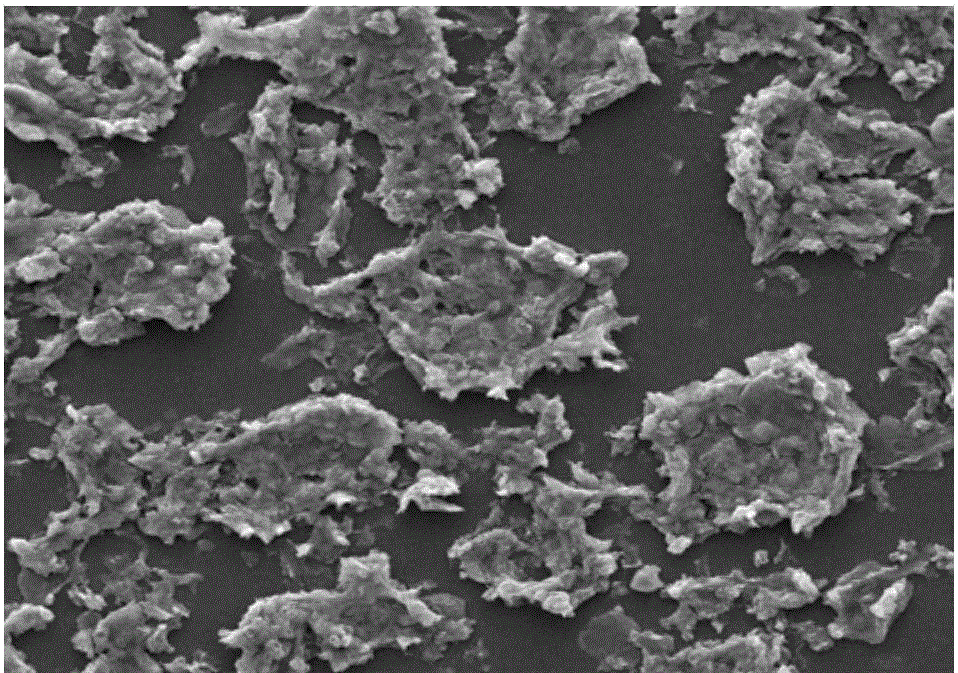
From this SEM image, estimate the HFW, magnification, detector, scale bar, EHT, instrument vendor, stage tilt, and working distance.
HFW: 298 µm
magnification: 1 K X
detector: ETD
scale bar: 100000 nm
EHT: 20 kV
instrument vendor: FEI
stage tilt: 0°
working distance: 11.2 mm